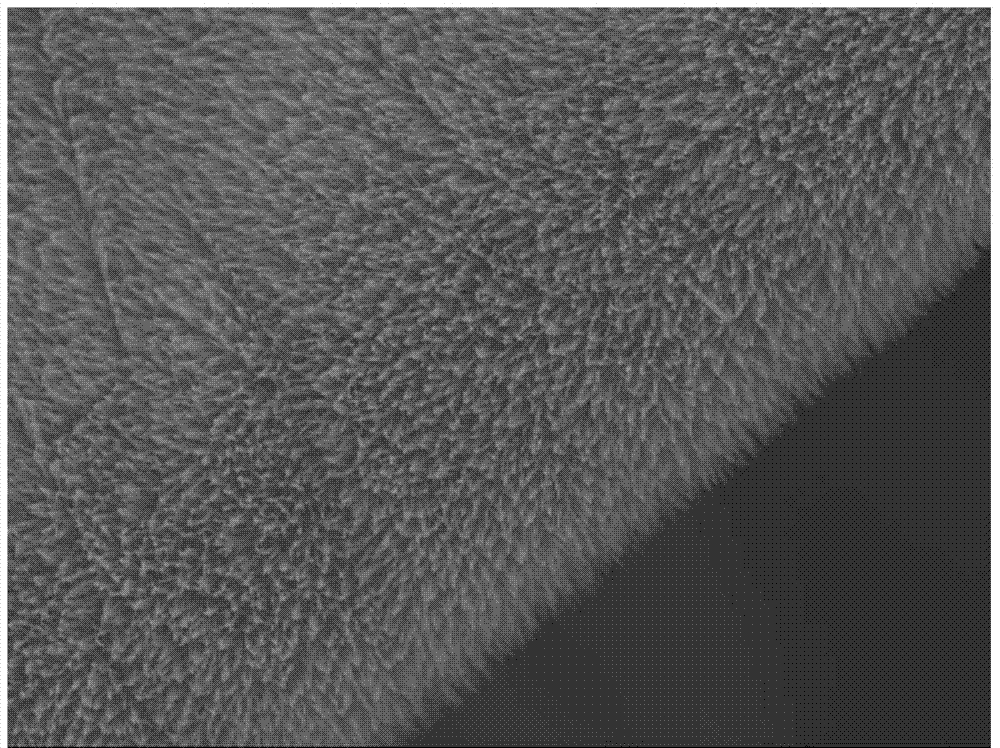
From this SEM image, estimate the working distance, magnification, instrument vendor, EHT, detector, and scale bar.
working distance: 10.2 mm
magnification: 5 K X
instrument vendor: JEOL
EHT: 15 kV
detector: SEI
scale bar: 1000 nm